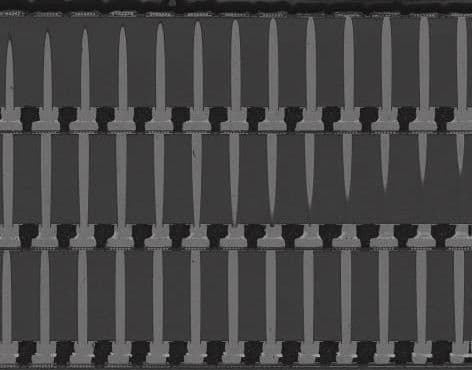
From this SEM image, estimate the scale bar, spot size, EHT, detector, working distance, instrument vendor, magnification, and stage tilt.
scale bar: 50000 nm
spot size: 5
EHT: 10 kV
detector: GAD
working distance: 4.4 mm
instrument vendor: FEI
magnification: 1.2 K X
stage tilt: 5°